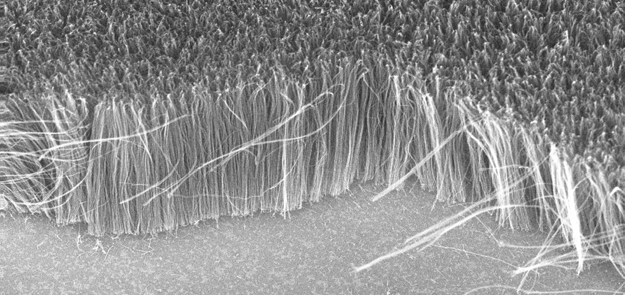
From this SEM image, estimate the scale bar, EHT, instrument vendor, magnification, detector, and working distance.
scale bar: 1000 nm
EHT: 20 kV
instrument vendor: Zeiss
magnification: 10 K X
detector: InLens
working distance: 5 mm